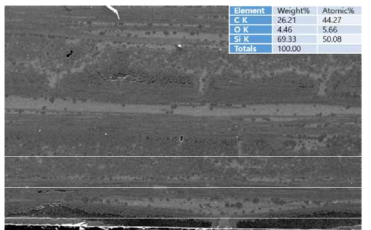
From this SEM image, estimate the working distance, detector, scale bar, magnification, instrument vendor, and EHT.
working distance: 11.9 mm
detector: SE(U)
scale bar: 500000 nm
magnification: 0.08 K X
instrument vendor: Hitachi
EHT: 20 kV